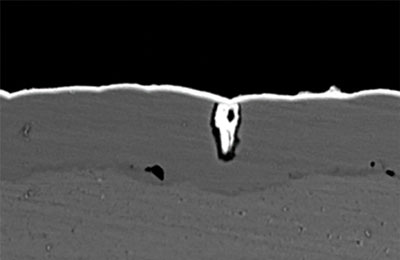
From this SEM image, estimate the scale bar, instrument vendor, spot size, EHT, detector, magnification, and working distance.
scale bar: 5000 nm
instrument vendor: JEOL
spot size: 50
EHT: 15 kV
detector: BEC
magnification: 3.5 K X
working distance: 7 mm